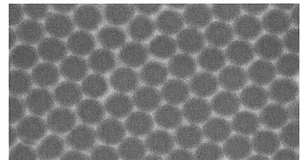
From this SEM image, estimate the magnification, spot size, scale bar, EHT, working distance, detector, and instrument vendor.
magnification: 240 K X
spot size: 3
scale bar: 200 nm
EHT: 10 kV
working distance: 5.1 mm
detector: TLD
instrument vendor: FEI